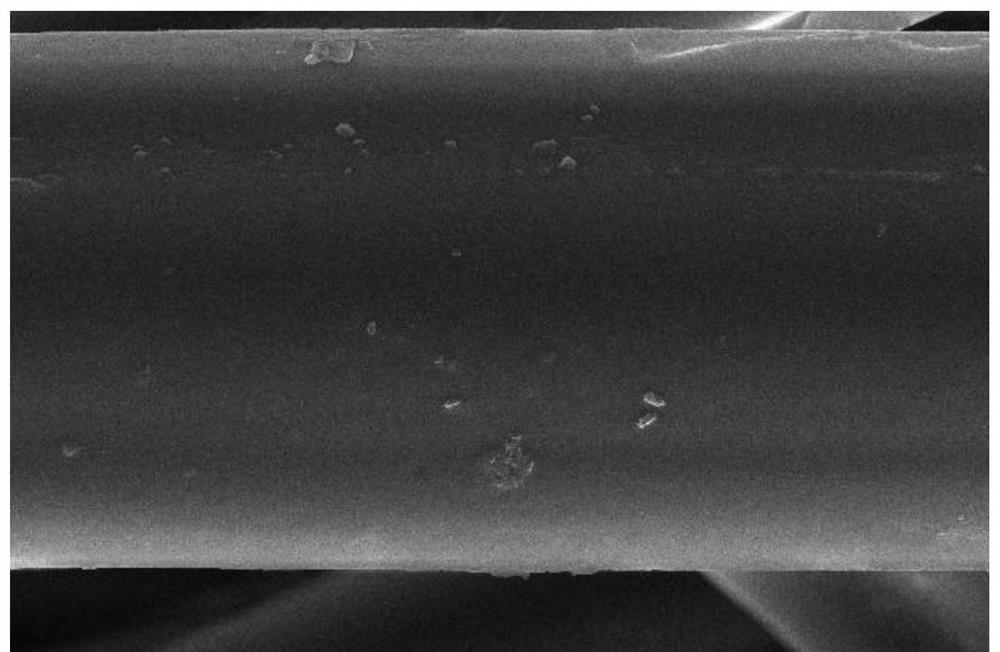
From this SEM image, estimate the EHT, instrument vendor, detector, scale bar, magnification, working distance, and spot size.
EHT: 20 kV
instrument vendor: FEI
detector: ETD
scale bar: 10000 nm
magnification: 10 K X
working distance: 11.9 mm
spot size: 6